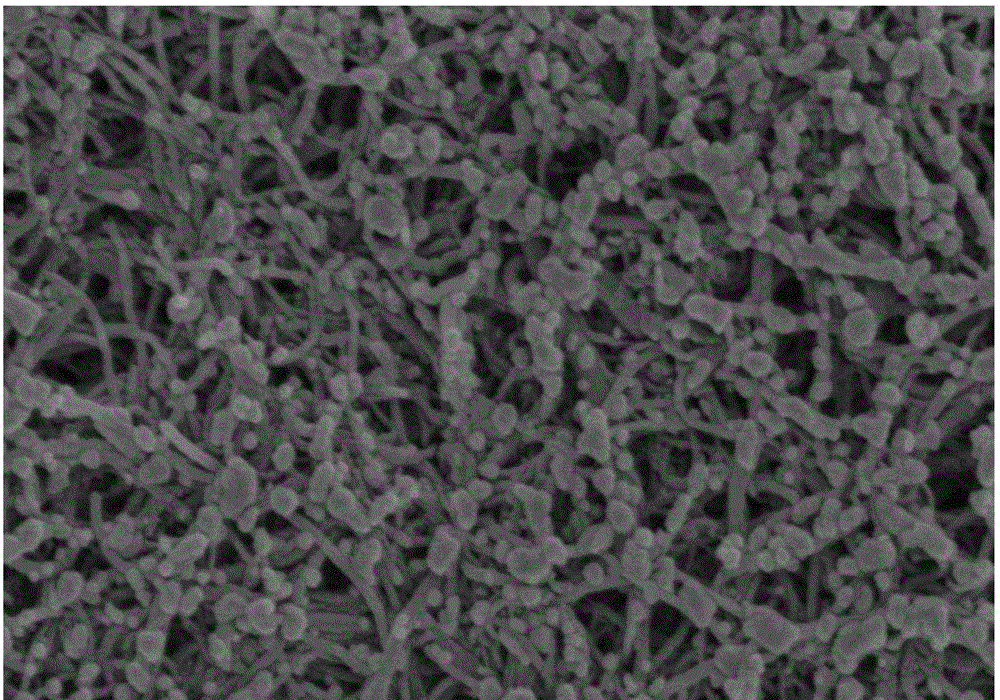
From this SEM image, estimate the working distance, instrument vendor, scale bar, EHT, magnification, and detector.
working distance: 8.7 mm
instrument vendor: Hitachi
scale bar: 1000 nm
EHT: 3 kV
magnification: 50 K X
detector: SE(UL)